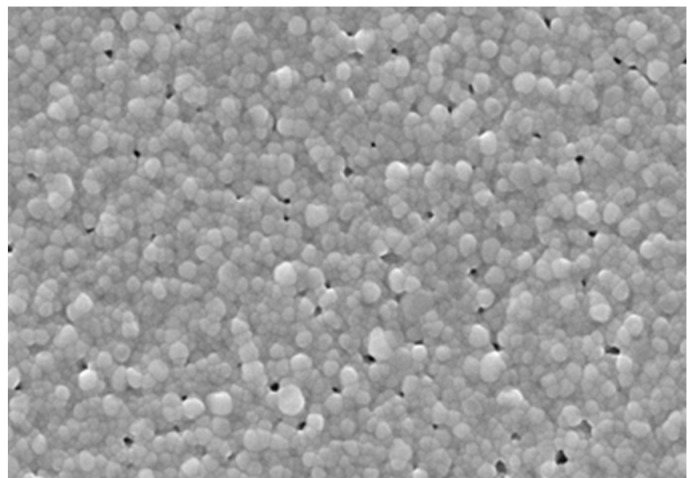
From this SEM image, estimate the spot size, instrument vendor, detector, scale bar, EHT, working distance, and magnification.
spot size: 3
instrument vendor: FEI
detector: SE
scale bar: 1000 nm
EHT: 15 kV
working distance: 4.7 mm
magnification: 50 K X